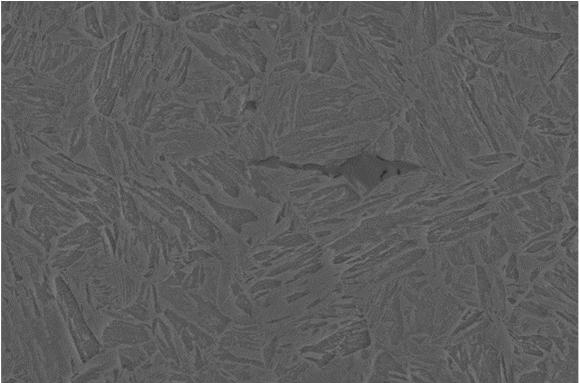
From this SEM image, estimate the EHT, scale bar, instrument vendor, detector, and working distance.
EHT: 15 kV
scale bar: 2000 nm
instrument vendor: Zeiss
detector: SE2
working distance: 8.5 mm